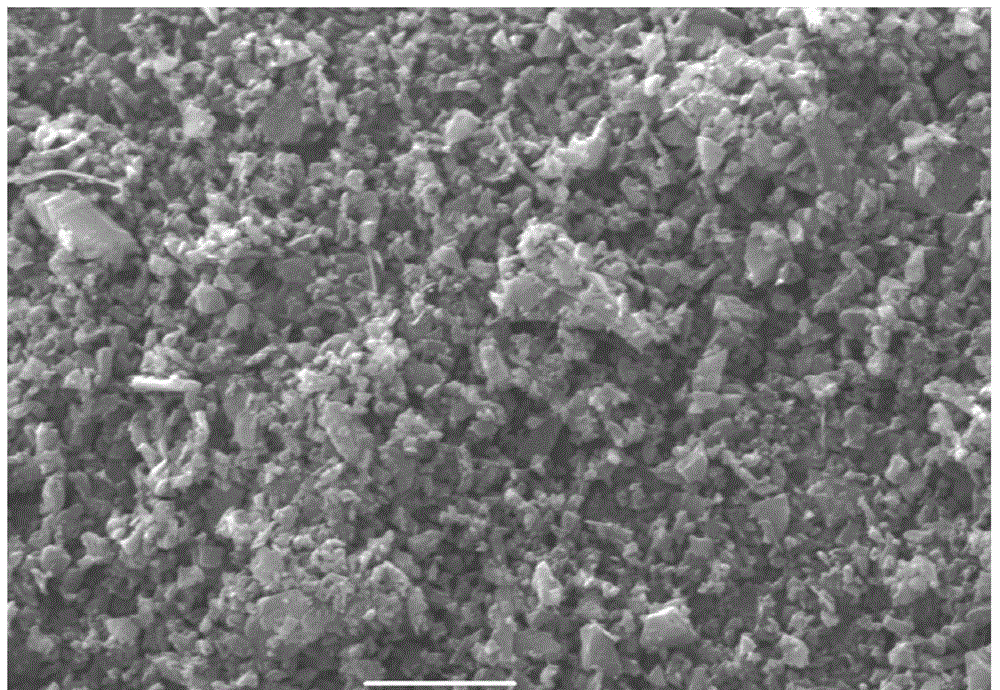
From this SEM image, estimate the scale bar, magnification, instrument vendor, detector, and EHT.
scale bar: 5000 nm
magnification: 4 K X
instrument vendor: JEOL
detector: SEI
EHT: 20 kV